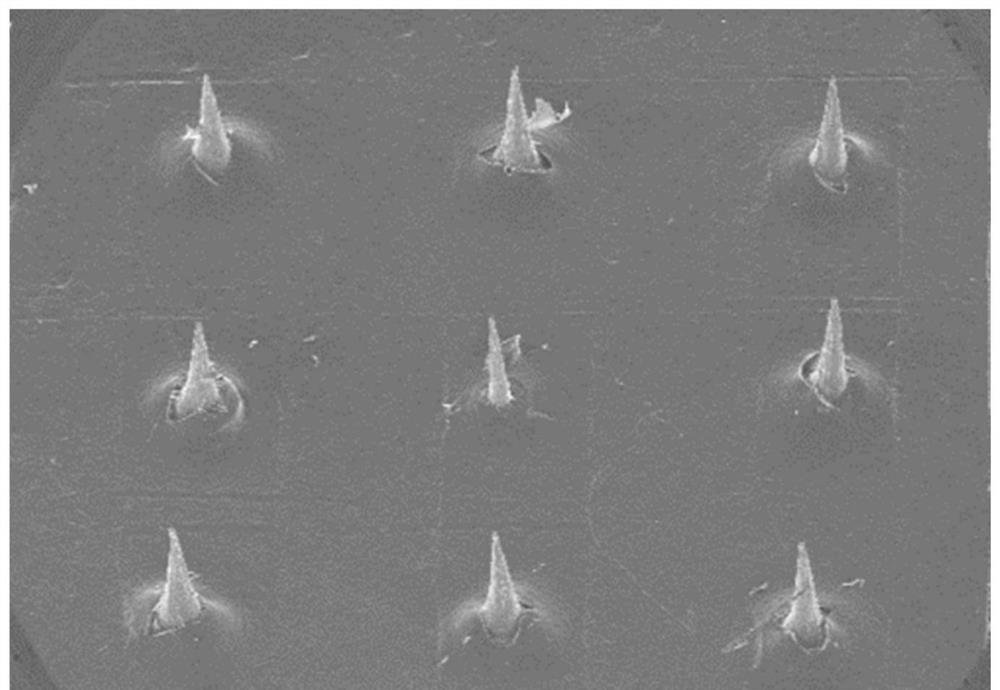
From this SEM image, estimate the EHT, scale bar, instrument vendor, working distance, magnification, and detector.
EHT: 15 kV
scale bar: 1000000 nm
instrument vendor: Hitachi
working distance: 13.8 mm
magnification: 0.03 K X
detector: SE(U)